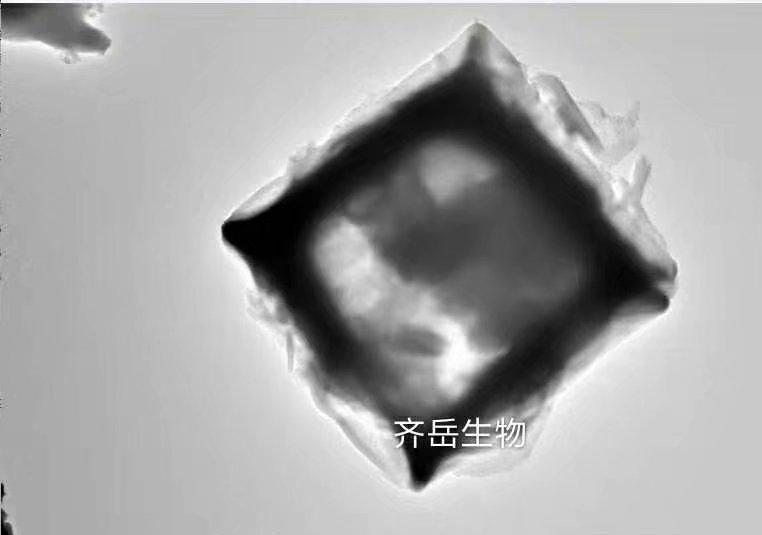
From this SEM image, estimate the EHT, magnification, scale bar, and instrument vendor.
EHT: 200 kV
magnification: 4 K X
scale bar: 2000 nm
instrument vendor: FEI